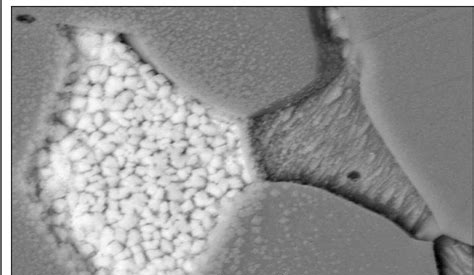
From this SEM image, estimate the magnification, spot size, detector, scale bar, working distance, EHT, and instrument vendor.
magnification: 8 K X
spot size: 3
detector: BSE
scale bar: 2000 nm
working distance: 5.4 mm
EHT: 20 kV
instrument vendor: FEI